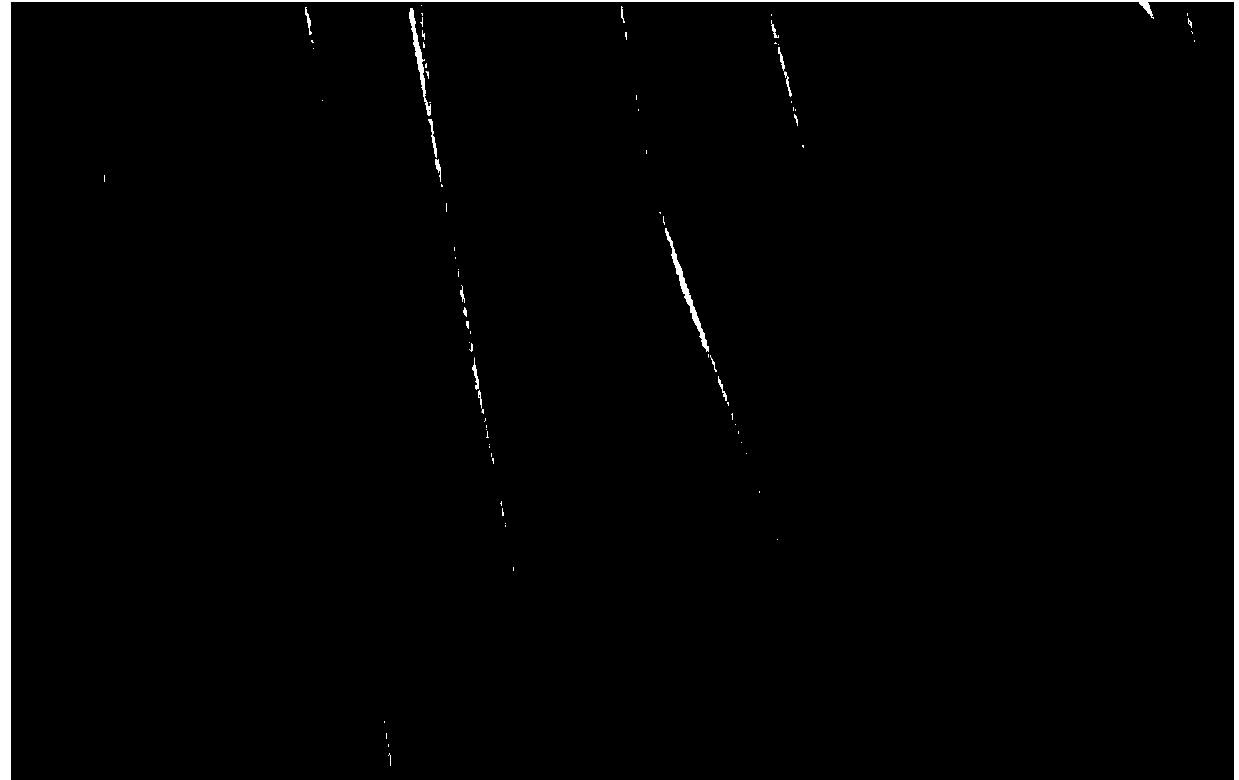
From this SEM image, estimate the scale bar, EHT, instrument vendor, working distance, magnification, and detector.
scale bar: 100000 nm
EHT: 1 kV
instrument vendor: Hitachi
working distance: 8.6 mm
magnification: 0.4 K X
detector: SE(U)